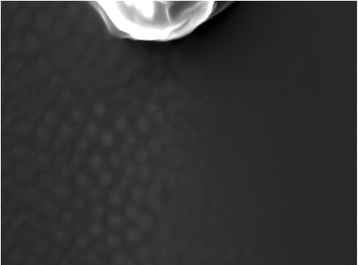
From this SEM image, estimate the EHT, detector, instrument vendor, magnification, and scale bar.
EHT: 30 kV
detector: SEI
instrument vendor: JEOL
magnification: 35 K X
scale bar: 100 nm